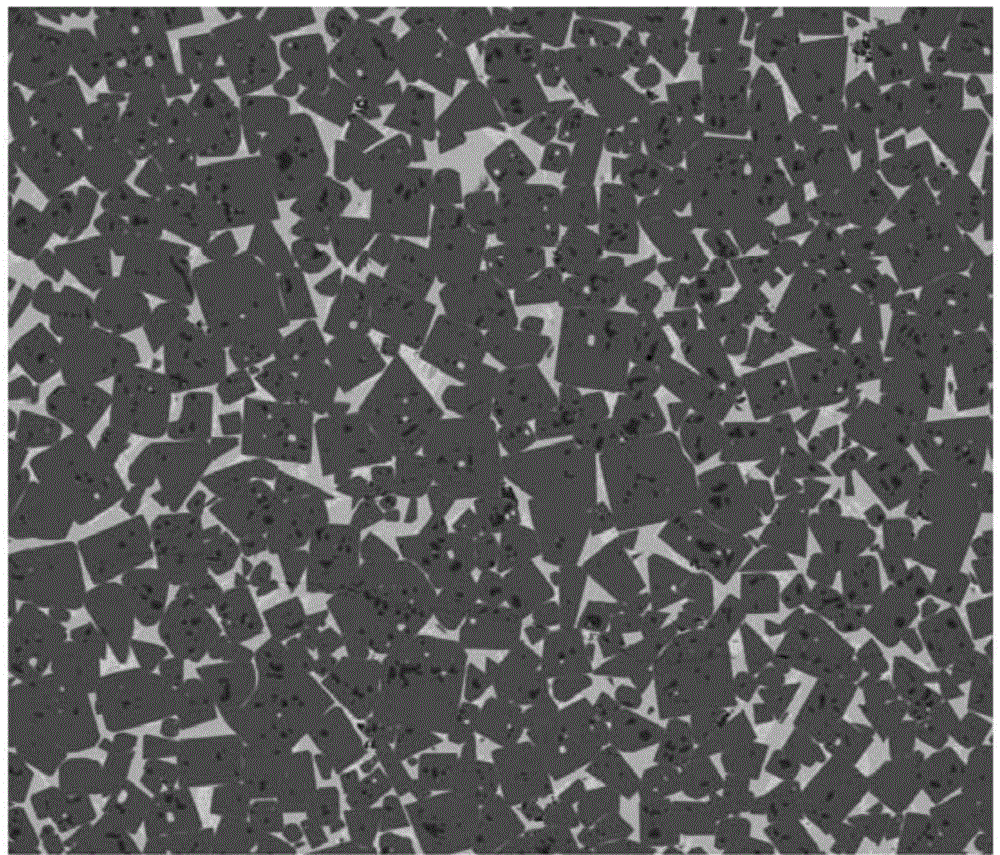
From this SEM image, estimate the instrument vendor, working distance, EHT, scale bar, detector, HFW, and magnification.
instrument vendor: FEI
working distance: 9.3 mm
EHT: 20 kV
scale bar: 500000 nm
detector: vCD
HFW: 1270 µm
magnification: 0.1 K X